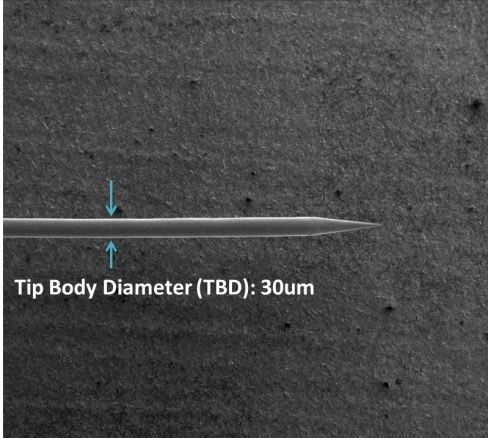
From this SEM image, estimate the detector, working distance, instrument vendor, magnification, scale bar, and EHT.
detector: ETD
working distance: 5 mm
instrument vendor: FEI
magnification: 0.08 K X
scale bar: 1000000 nm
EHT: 5 kV